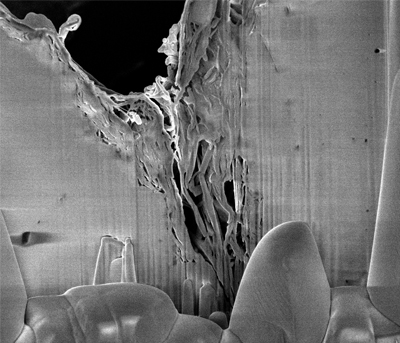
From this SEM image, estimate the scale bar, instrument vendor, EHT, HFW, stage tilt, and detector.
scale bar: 5000 nm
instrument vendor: FEI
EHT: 2 kV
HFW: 10.2 µm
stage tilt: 52°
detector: TLD